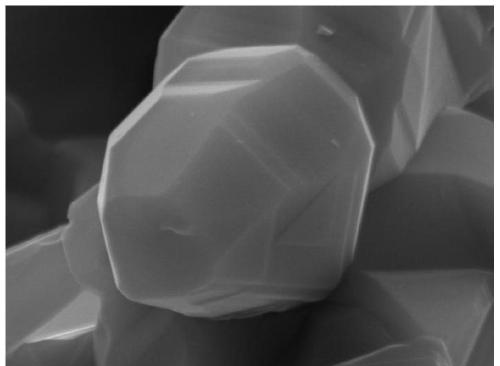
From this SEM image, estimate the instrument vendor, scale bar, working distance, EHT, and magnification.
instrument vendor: Tescan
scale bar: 1000 nm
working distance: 15.61 mm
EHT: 20 kV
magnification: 50 K X